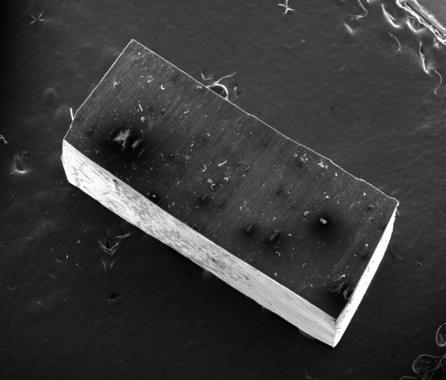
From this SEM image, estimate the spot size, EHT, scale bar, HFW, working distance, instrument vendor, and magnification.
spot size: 3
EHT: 20 kV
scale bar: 1000000 nm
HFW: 3450 µm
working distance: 11.2 mm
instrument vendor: FEI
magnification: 0.043 K X